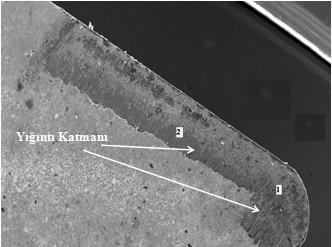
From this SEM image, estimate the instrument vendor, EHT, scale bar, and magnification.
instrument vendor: JEOL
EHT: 20 kV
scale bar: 500000 nm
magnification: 0.05 K X